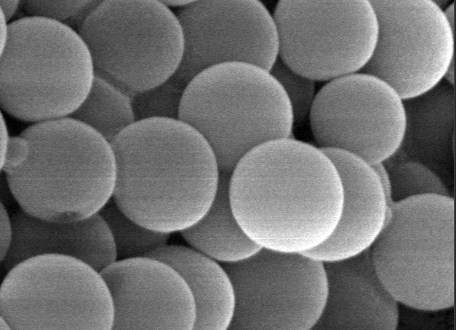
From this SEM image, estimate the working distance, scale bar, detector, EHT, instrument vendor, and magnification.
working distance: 7.5 mm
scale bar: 100 nm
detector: SEM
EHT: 15 kV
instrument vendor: JEOL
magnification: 50 K X